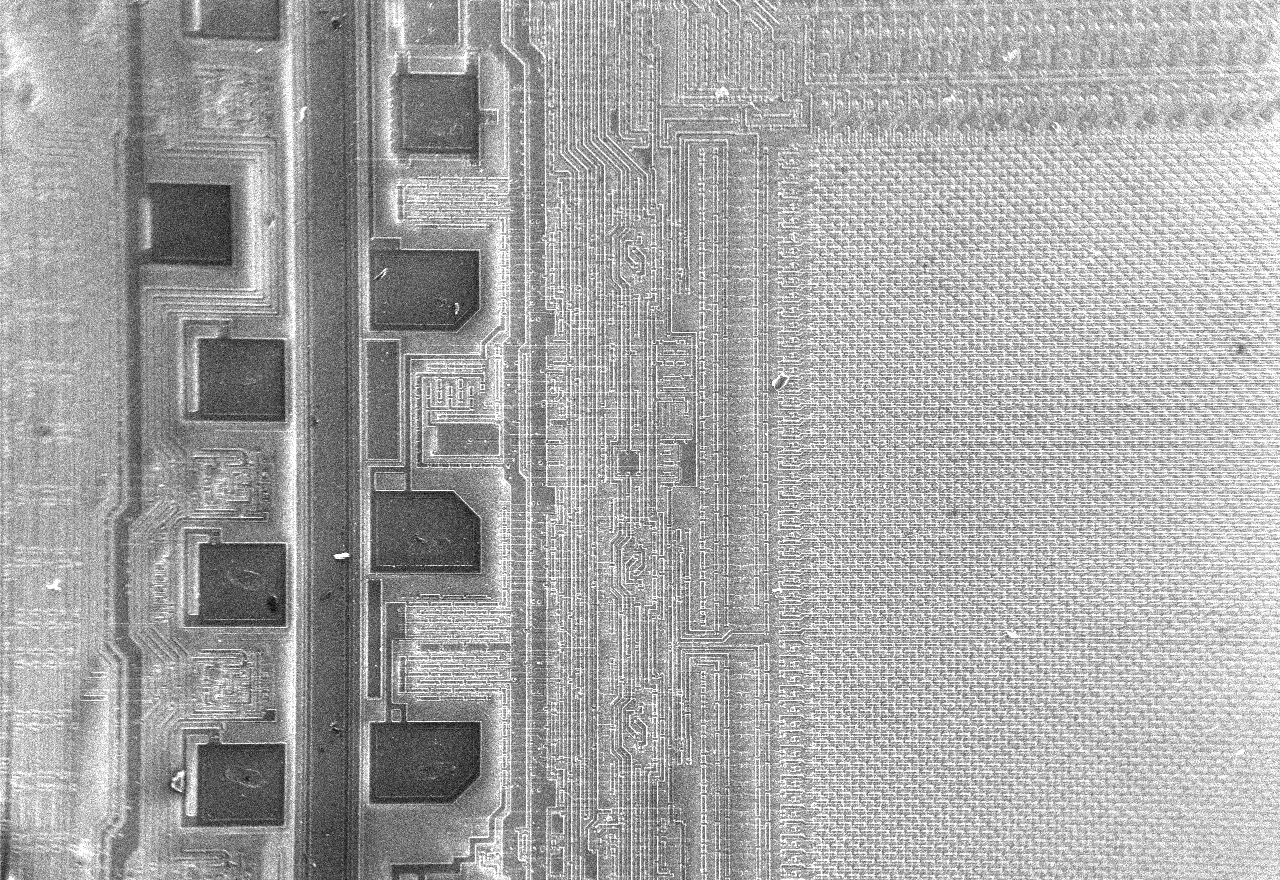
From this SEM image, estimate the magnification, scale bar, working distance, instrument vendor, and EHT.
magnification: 0.05 K X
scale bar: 200000 nm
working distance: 8.3 mm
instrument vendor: JEOL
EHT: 15 kV